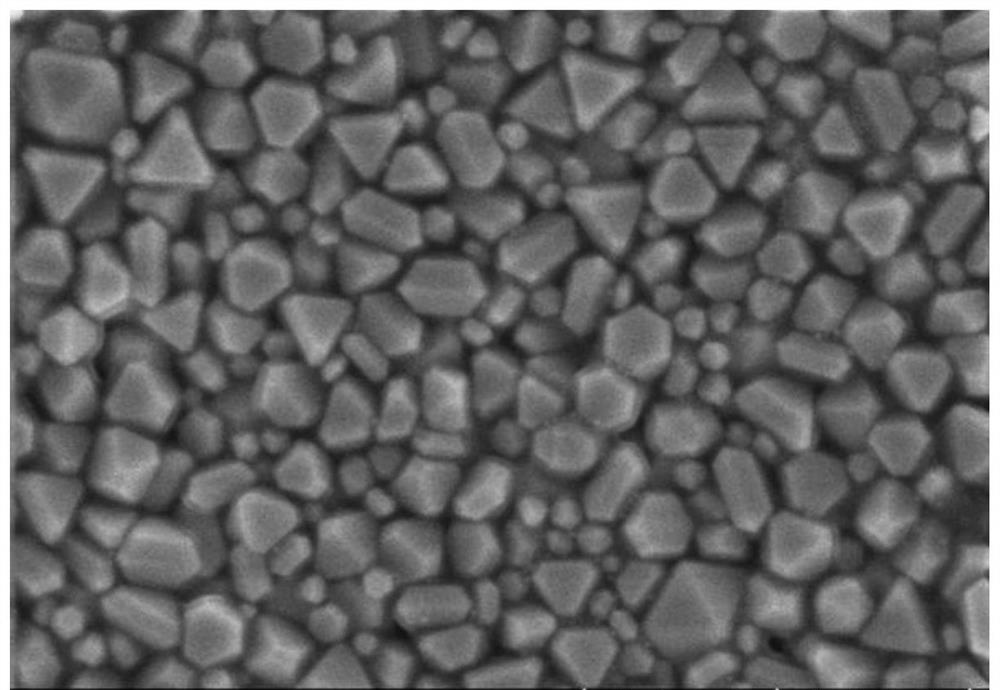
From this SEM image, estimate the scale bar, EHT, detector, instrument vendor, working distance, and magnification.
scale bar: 1000 nm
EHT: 5 kV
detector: SE(UL)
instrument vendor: Hitachi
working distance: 8.4 mm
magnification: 50 K X